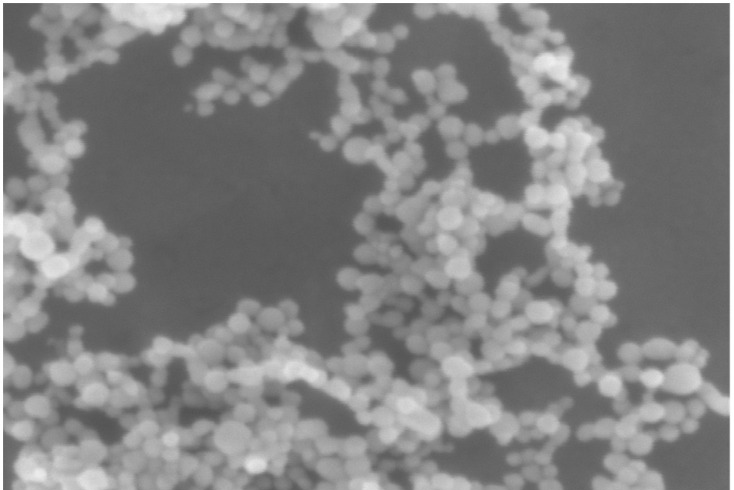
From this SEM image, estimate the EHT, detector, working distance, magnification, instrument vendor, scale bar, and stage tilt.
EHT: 5 kV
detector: InLens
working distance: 5 mm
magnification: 200 K X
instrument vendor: Zeiss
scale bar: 200 nm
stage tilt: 8.1°